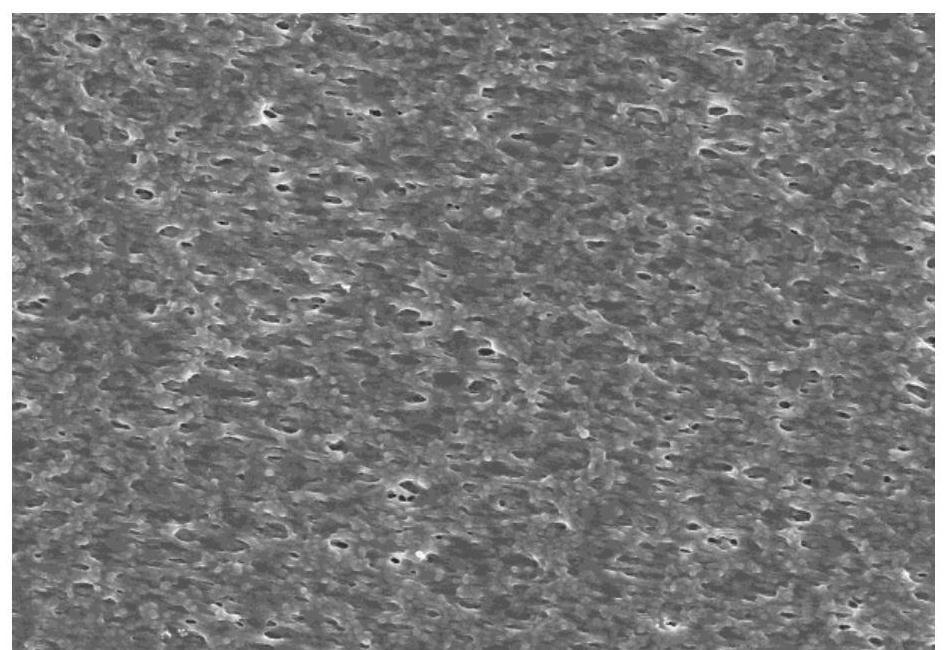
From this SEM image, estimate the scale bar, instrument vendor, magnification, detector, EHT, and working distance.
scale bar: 10000 nm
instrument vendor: Hitachi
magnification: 5 K X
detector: SE(U)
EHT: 5 kV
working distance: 8.9 mm